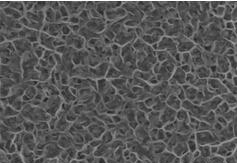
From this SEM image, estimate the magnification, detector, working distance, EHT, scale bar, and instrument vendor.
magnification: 40 K X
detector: SE(M)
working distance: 8.1 mm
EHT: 5 kV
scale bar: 1000 nm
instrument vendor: Hitachi